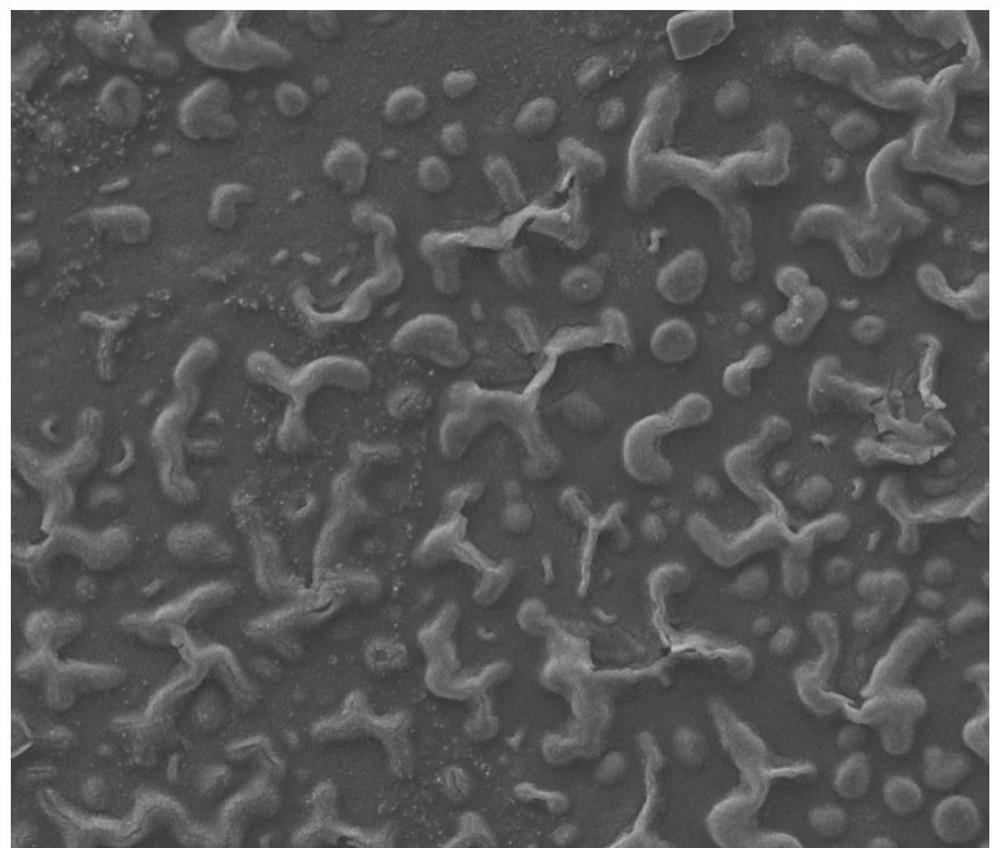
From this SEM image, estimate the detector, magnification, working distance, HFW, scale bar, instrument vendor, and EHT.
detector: ETD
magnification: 2 K X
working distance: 6.2 mm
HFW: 135 µm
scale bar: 40000 nm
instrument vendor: FEI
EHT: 10 kV